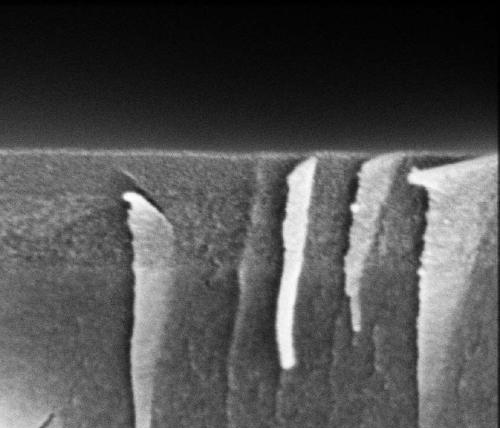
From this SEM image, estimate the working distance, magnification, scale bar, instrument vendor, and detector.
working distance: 5 mm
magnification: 120 K X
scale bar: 500 nm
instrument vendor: FEI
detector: TLD